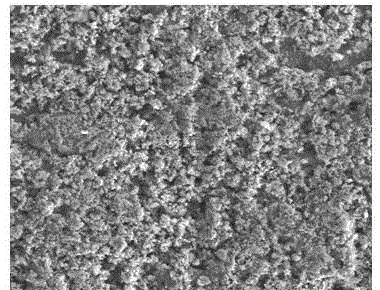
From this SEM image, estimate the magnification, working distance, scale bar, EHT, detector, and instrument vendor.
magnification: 5 K X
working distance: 4.9 mm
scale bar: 10000 nm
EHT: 15 kV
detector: ETD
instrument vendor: FEI